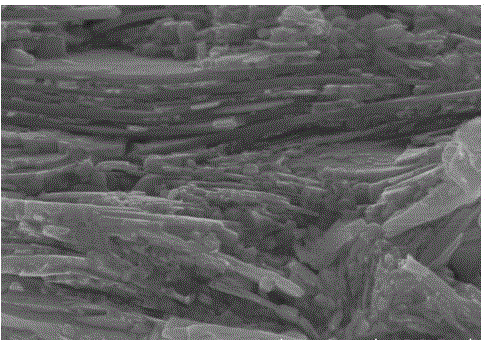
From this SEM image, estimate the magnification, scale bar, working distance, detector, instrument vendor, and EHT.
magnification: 50 K X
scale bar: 1000 nm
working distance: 9.2 mm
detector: SE(U)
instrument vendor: Hitachi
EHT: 10 kV